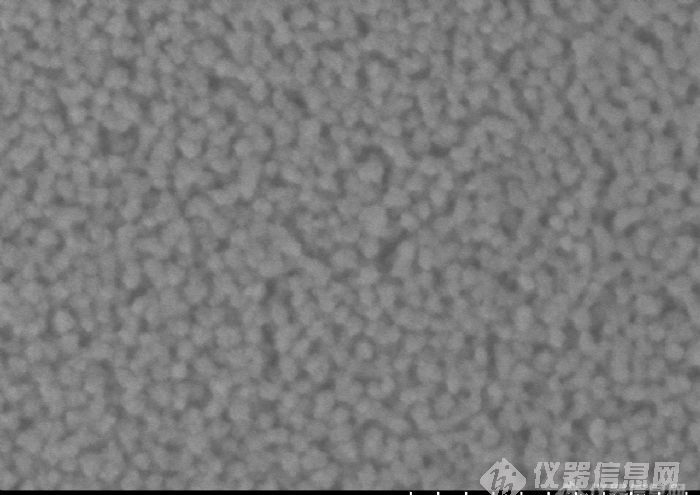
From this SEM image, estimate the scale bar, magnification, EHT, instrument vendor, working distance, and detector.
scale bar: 200 nm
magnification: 250 K X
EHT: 1 kV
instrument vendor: Hitachi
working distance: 1.8 mm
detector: SE(M,LA0)